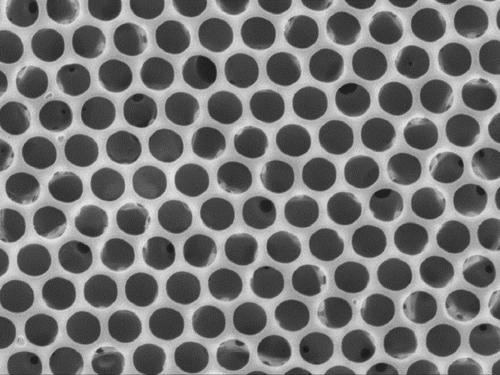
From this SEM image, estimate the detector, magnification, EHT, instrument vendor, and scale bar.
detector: SEI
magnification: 3 K X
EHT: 15 kV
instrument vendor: JEOL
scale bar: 1000 nm